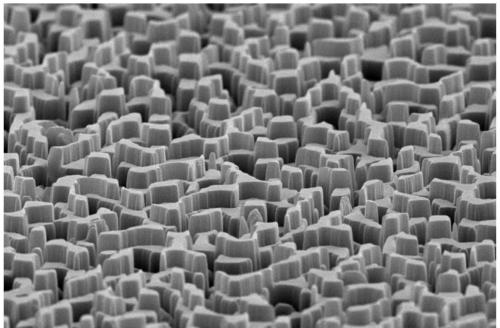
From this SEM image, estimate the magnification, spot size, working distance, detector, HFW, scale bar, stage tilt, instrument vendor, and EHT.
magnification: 10 K X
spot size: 3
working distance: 12.8 mm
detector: ETD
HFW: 12.7 µm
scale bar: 5000 nm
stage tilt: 8°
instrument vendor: FEI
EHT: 10 kV